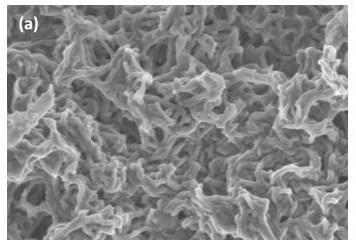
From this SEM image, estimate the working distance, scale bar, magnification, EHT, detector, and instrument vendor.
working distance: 8.1 mm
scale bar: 1000 nm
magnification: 30 K X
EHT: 2 kV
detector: SE(M)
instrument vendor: Hitachi